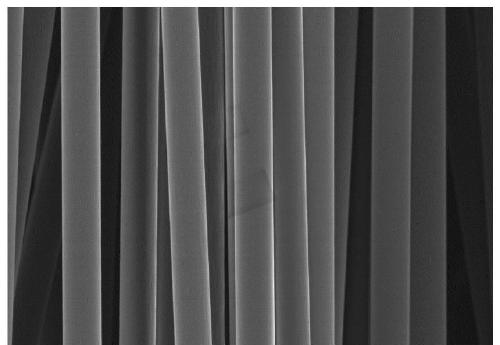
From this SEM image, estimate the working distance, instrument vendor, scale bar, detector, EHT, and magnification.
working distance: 10.5 mm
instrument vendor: Hitachi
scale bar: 30000 nm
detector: SE(M)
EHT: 5 kV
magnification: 1.8 K X